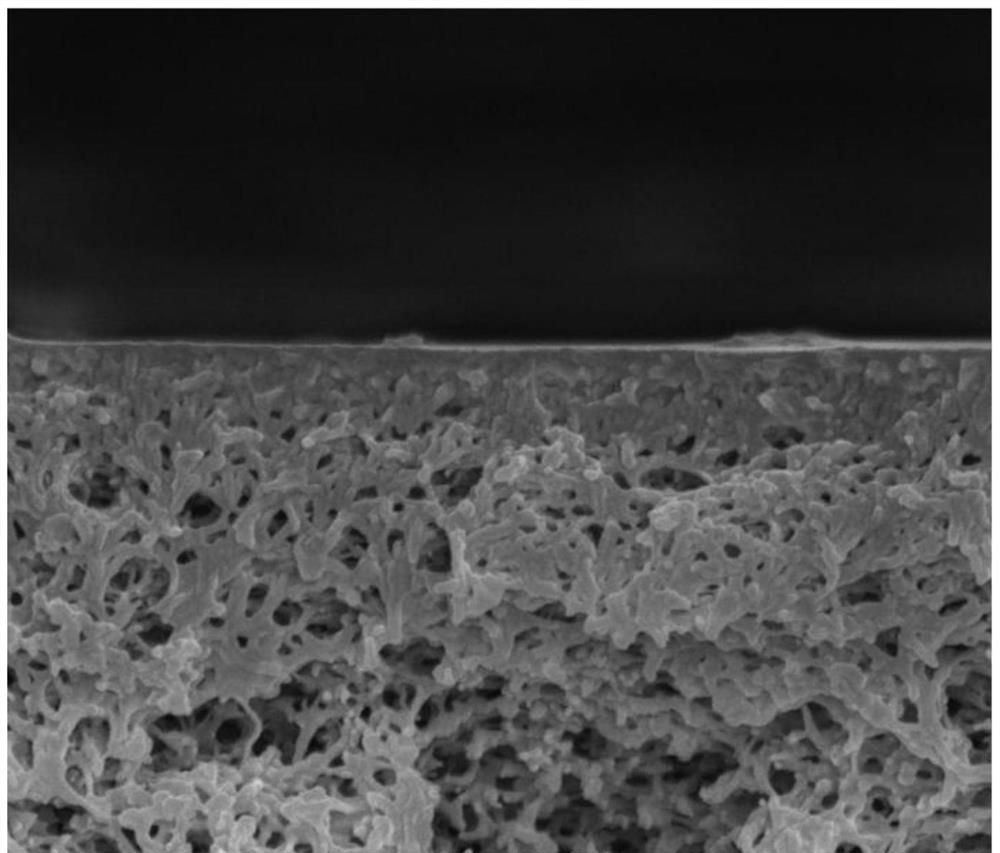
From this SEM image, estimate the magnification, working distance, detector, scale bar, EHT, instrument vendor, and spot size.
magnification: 80 K X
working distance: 4.9 mm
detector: TLD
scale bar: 1000 nm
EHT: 5 kV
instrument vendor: FEI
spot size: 3.5